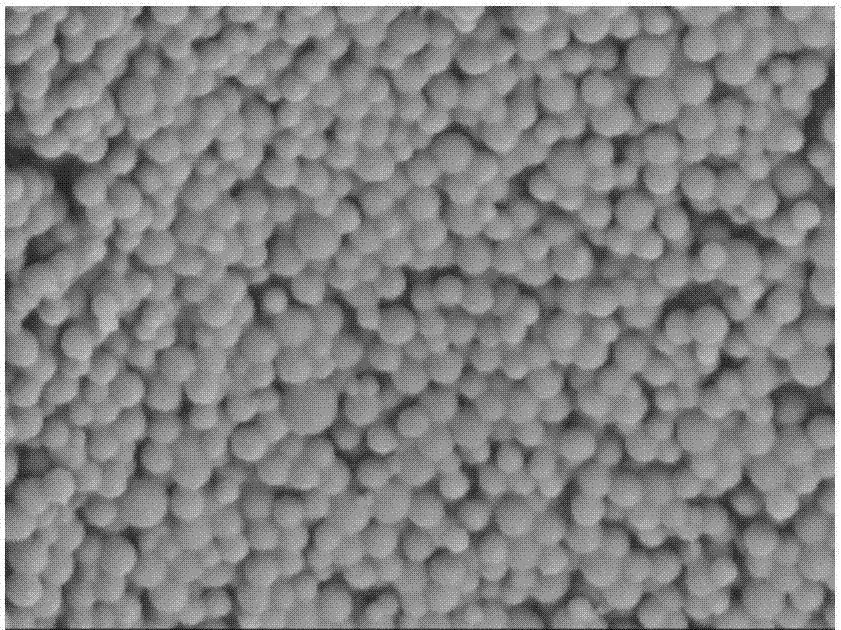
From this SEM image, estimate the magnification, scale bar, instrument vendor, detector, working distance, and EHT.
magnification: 20 K X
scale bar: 1000 nm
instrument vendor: JEOL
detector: SEI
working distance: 23.2 mm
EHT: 3 kV